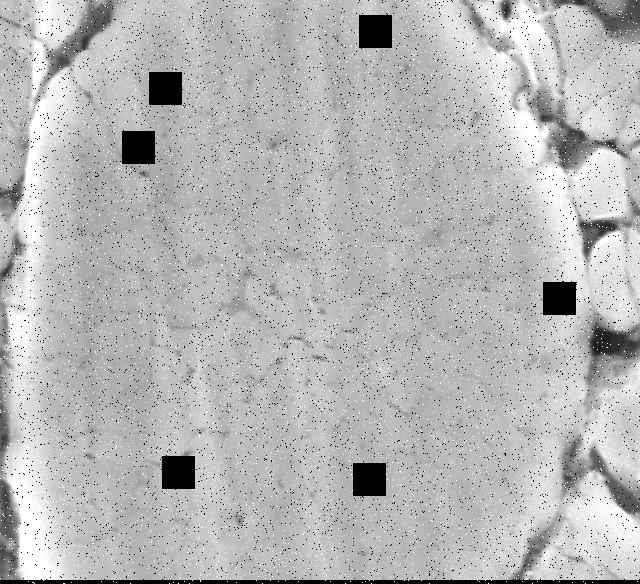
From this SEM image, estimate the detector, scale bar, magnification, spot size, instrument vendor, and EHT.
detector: SEI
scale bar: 1000 nm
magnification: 10 K X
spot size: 45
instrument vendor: JEOL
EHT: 15 kV